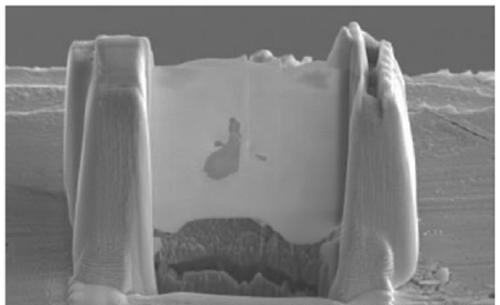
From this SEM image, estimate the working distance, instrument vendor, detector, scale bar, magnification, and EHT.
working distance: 5 mm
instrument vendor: Hitachi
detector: SE(L)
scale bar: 10000 nm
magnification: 5 K X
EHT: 10 kV